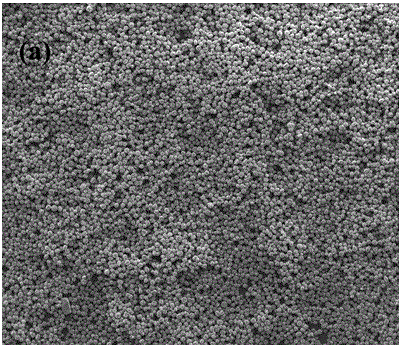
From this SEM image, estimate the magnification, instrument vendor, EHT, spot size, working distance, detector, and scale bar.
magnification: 0.2 K X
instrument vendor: FEI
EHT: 30 kV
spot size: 3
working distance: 9.8 mm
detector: ETD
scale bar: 500000 nm